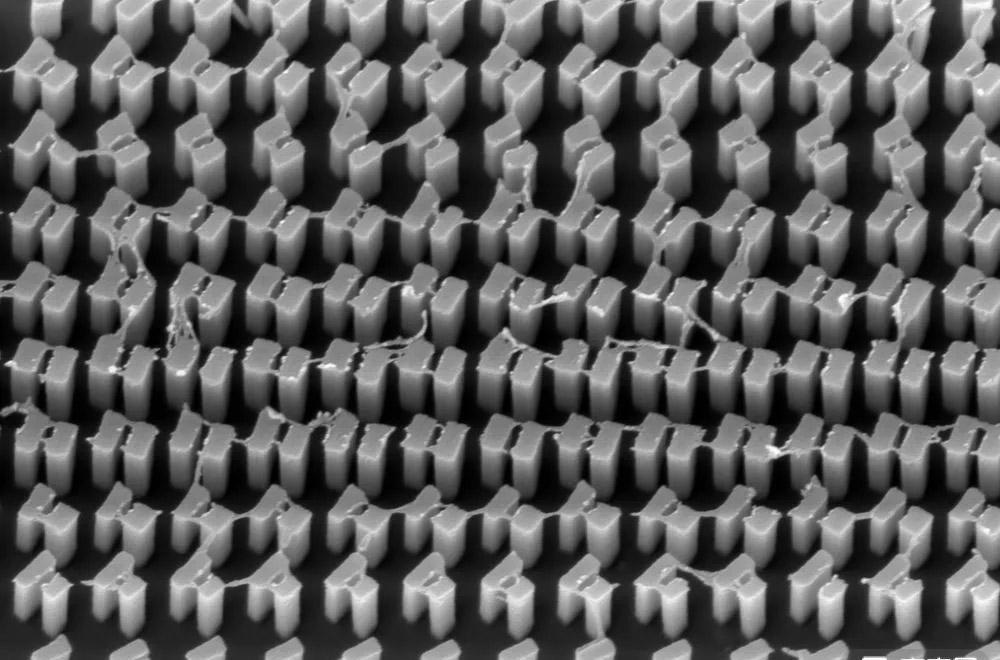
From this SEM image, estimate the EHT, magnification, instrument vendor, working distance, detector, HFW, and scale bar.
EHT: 5 kV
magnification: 40 K X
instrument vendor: FEI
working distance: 5 mm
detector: TLD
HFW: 5.18 µm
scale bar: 2000 nm